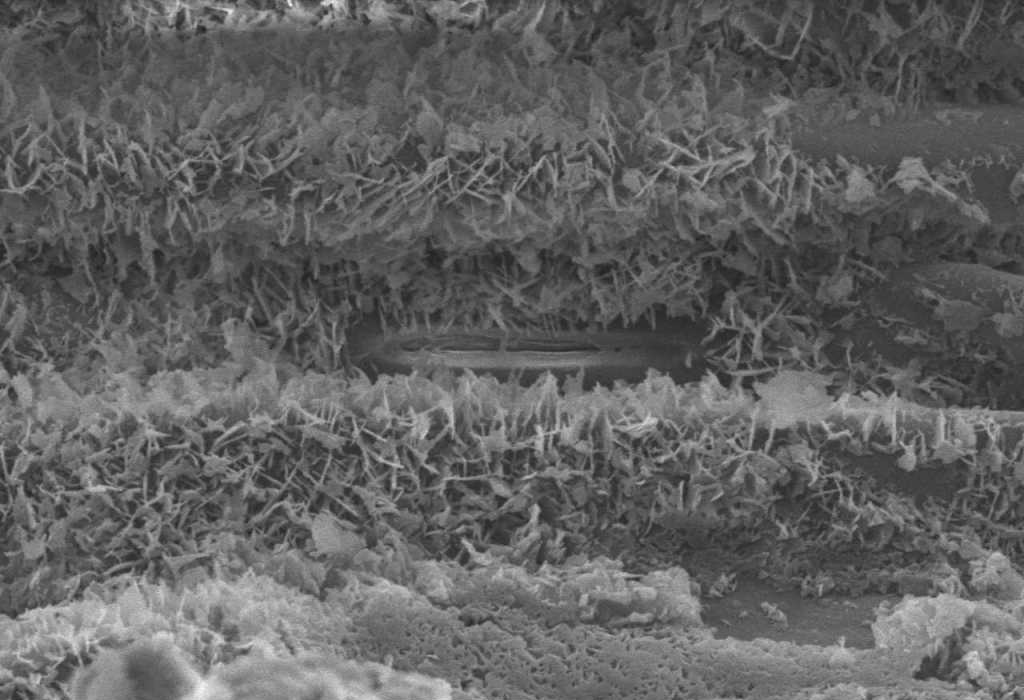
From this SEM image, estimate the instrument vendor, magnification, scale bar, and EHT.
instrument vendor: JEOL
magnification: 3.7 K X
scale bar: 5000 nm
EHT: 9 kV